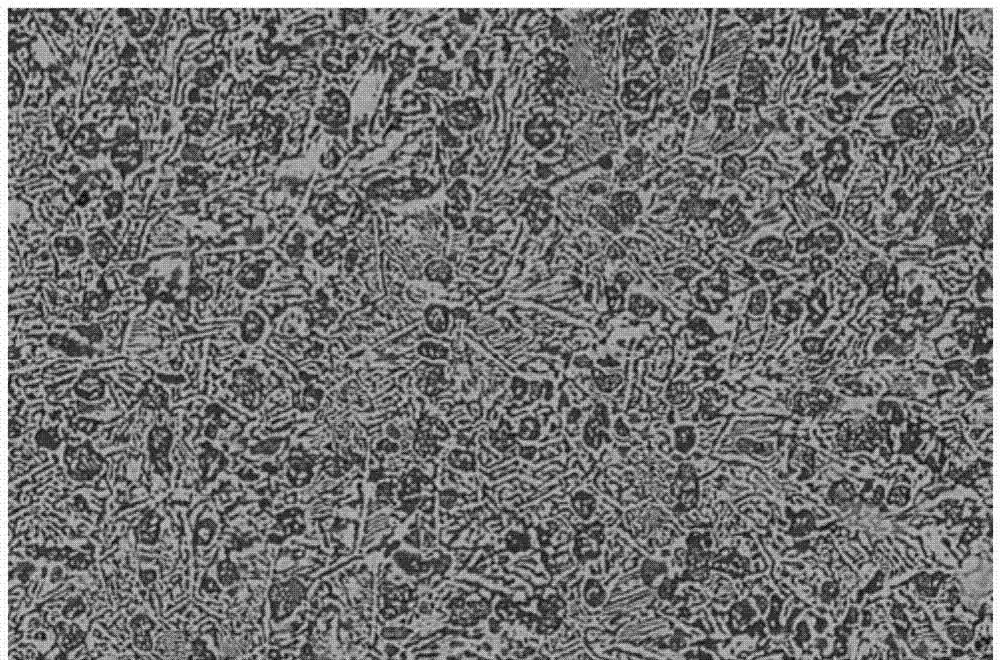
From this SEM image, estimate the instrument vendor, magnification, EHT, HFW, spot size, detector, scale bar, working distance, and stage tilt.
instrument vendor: FEI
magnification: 2 K X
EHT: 20 kV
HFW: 207 µm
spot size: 3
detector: BSED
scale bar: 50000 nm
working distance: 12 mm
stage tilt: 0°